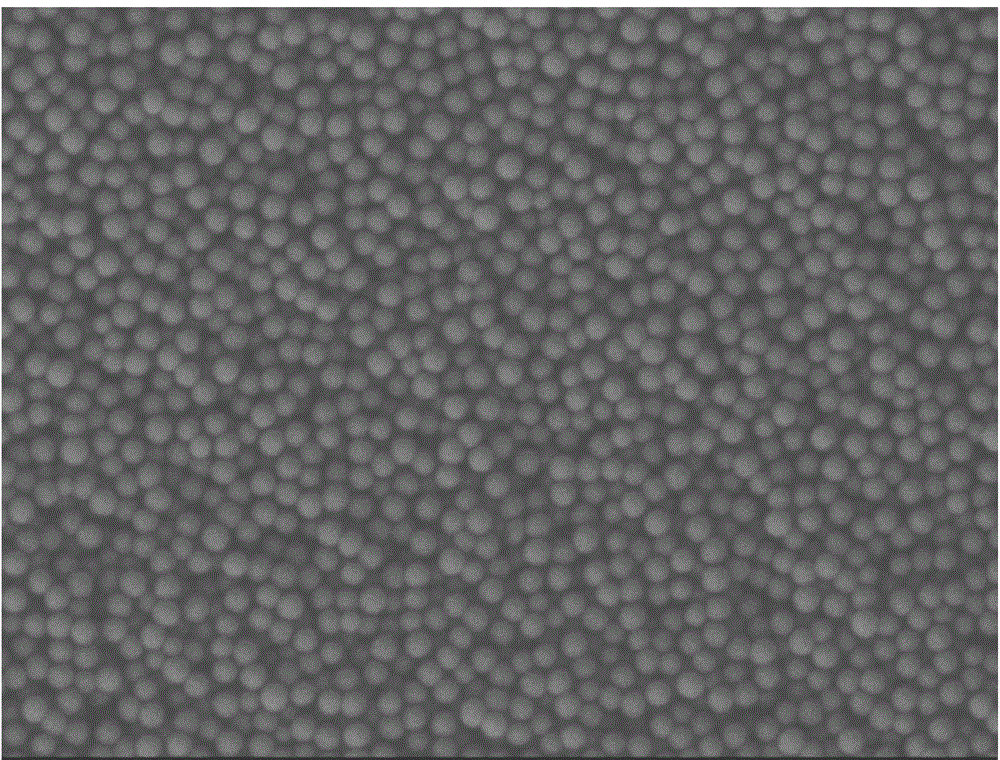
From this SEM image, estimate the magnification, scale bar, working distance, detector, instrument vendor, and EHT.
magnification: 30 K X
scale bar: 100 nm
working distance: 6.9 mm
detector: LEI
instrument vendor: JEOL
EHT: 3 kV